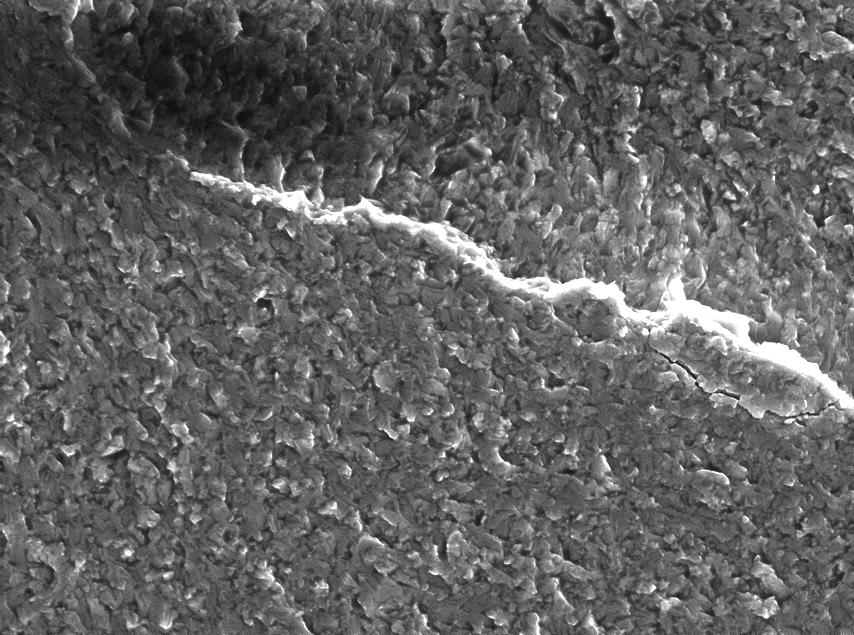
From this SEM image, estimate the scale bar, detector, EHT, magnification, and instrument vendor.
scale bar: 50000 nm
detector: SE Detector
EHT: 20 kV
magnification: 2 K X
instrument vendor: Tescan